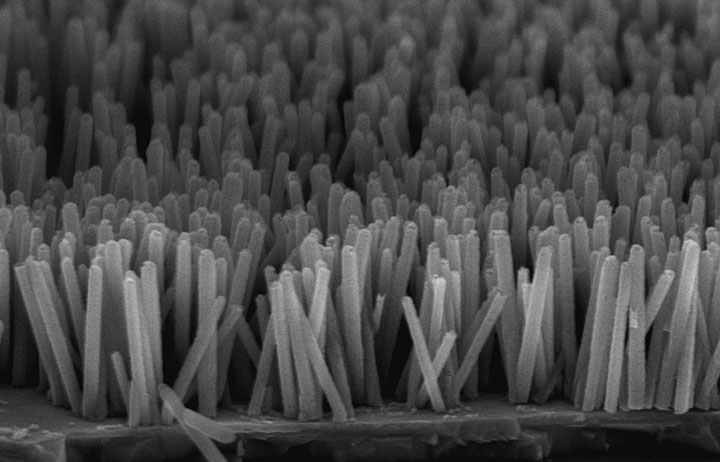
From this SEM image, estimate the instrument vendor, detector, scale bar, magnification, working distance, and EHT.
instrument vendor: Hitachi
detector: SE(M,LA0)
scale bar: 1000 nm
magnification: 50 K X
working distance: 7.5 mm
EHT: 5 kV